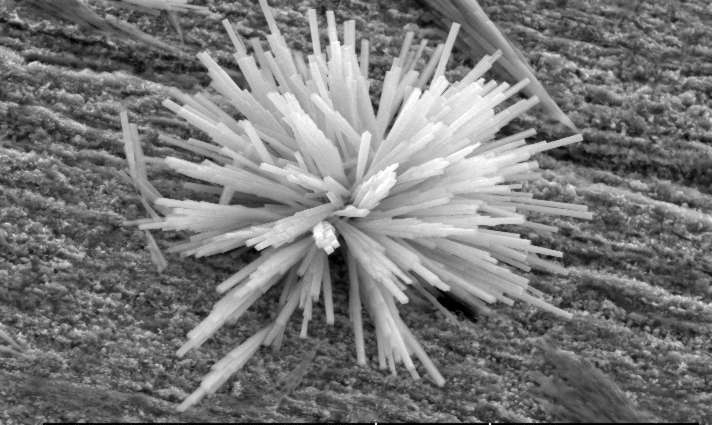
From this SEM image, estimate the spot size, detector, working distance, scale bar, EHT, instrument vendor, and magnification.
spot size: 3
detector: GSE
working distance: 12.7 mm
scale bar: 10000 nm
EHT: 20 kV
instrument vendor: FEI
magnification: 2 K X